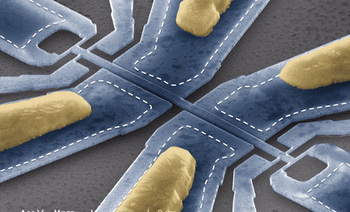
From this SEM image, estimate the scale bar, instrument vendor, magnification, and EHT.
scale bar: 2000 nm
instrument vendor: FEI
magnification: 10 K X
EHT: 15 kV